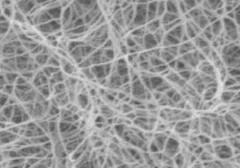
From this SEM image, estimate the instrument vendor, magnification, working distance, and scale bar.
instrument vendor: JEOL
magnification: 50 K X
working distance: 4.1 mm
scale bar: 100 nm